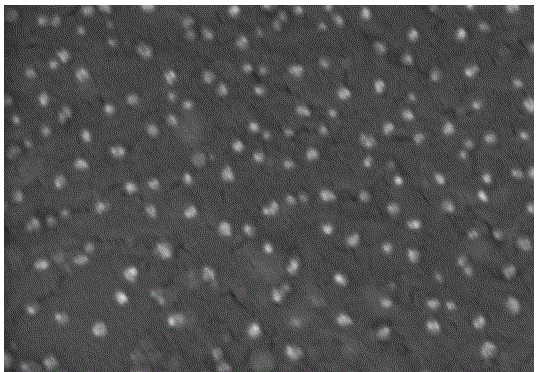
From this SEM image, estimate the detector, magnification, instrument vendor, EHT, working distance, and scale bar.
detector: SE(M)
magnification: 80 K X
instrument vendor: Hitachi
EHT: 3 kV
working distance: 9.5 mm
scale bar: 500 nm